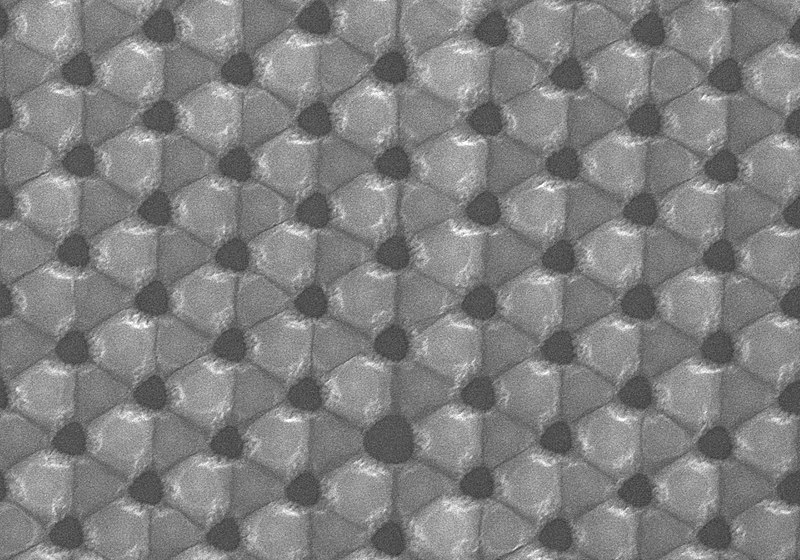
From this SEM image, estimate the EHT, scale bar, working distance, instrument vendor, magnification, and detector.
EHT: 15 kV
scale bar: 3000 nm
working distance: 6.1 mm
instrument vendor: Hitachi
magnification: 15 K X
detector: SE(U)